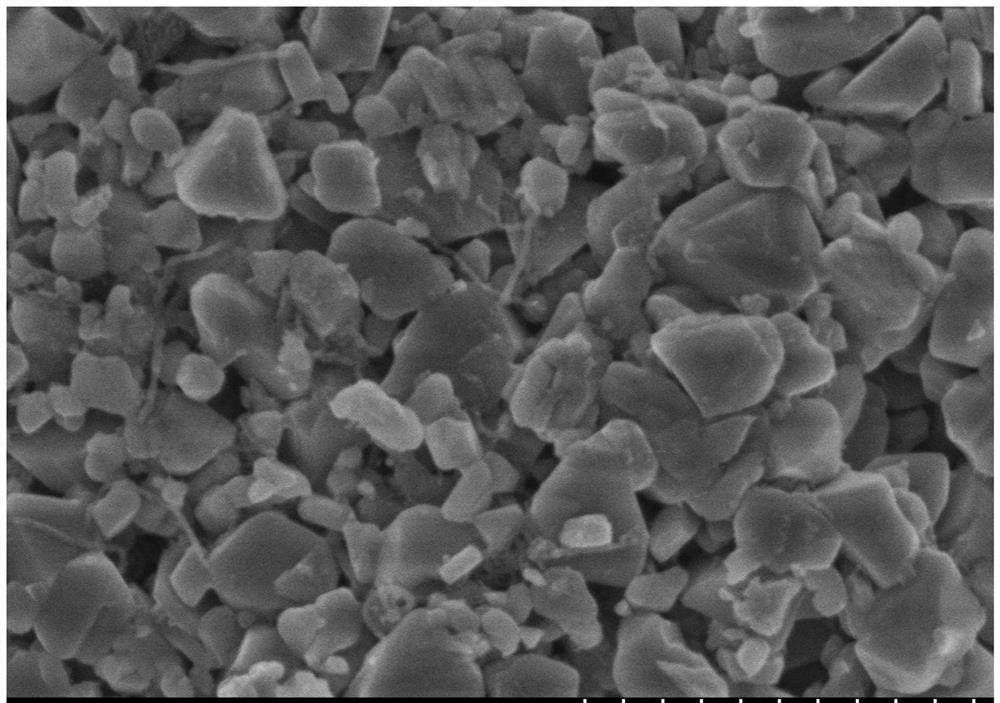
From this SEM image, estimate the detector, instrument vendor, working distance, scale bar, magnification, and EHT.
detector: SE(M)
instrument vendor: Hitachi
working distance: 8 mm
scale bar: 1000 nm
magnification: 50 K X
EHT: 3 kV